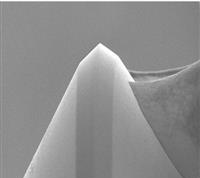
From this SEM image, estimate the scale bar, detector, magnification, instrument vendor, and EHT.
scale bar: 100000 nm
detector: BED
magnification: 0.3 K X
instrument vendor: JEOL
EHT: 20 kV